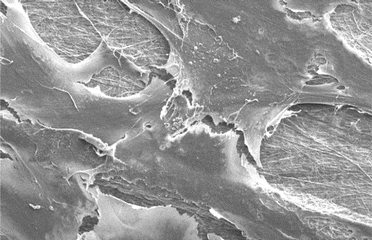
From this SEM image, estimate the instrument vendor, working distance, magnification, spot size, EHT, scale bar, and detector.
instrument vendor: FEI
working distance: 5 mm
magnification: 2 K X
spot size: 2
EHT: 5 kV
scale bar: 20000 nm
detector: SE